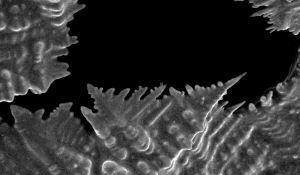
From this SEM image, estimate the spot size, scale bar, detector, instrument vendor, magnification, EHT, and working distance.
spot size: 2.5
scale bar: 50000 nm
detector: SE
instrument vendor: FEI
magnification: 0.726 K X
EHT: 30 kV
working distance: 7.3 mm